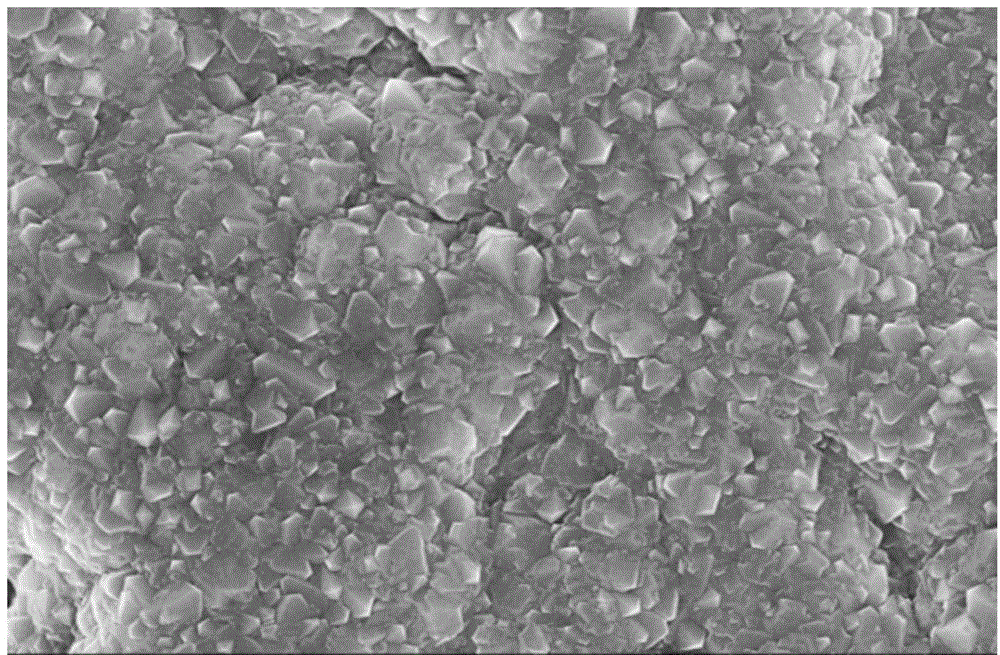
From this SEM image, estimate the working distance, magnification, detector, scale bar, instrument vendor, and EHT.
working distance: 8.9 mm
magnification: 10 K X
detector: SE(M)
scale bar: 5000 nm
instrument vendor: Hitachi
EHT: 10 kV